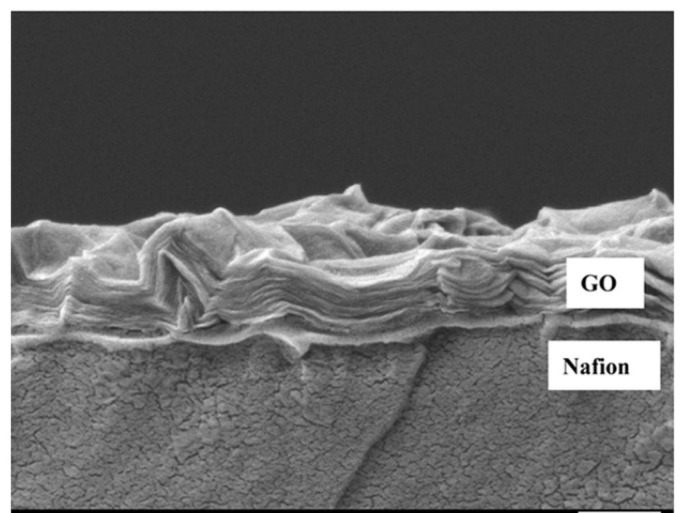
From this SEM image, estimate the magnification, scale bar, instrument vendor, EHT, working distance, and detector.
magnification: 15 K X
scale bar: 1000 nm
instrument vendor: JEOL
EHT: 3 kV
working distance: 15.1 mm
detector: LEI